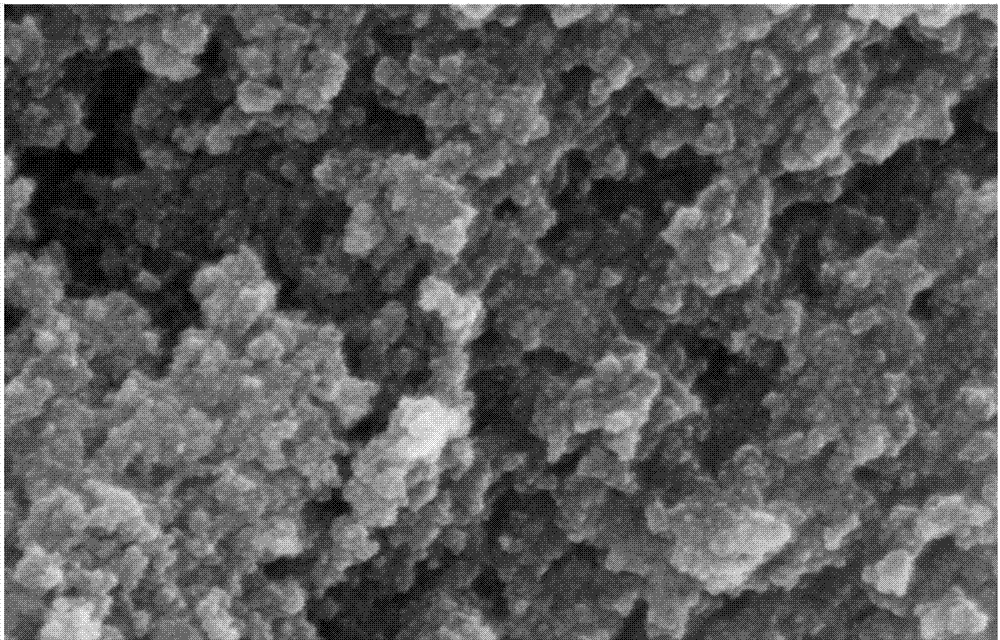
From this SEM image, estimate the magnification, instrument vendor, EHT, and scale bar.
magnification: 100 K X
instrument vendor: Hitachi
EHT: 5 kV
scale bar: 500 nm